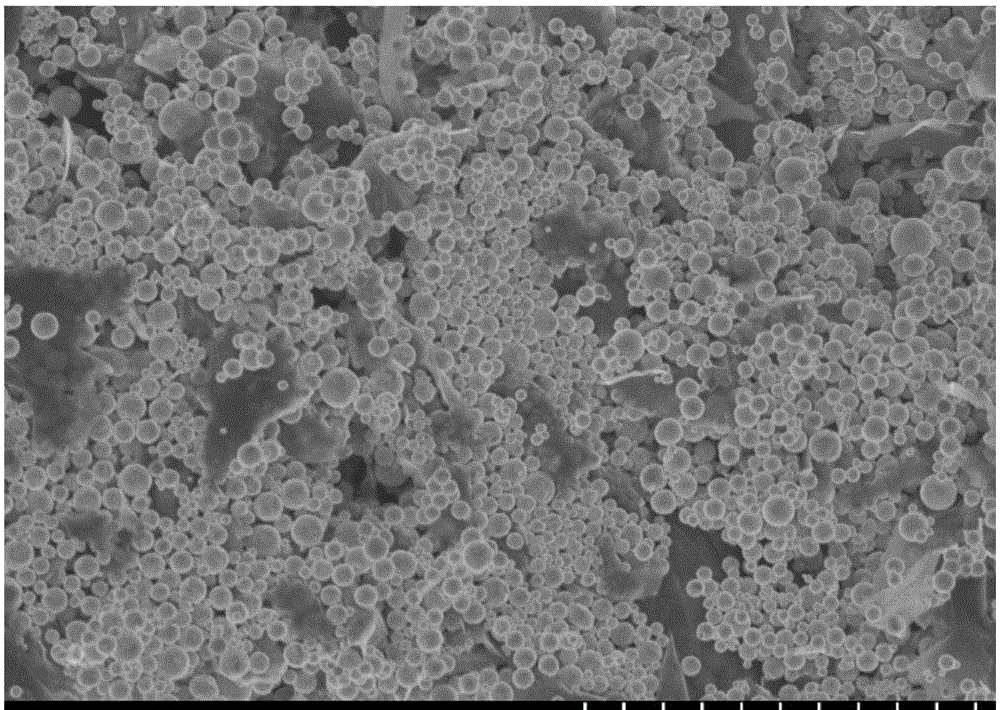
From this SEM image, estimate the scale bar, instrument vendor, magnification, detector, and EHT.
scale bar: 10000 nm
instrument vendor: Hitachi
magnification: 5 K X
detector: SE(U)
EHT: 4 kV